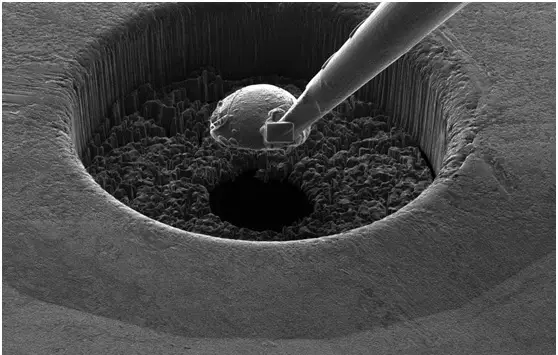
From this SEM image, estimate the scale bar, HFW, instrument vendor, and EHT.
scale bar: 30000 nm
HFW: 59.2 µm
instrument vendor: FEI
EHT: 30 kV